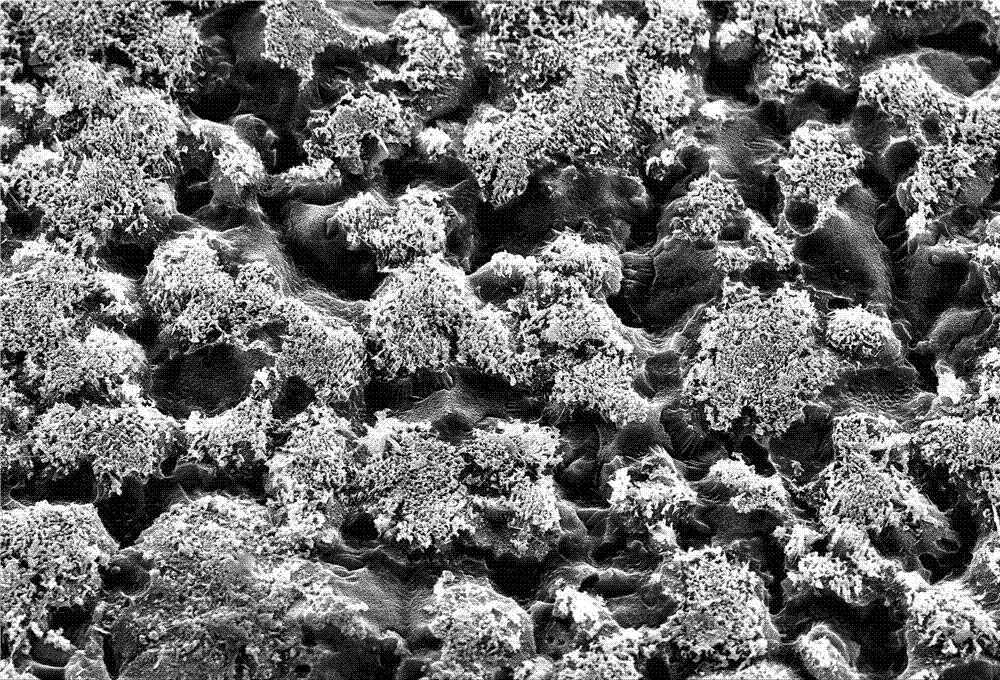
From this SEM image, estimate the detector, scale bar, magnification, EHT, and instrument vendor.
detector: SEI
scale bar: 10000 nm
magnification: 1 K X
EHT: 30 kV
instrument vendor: JEOL